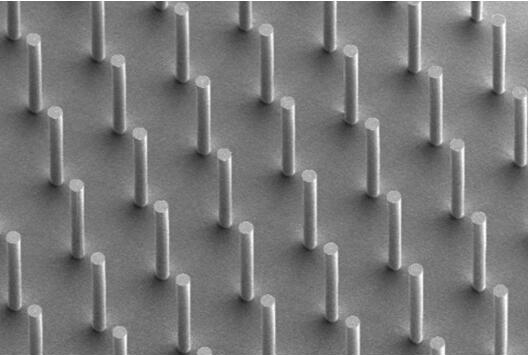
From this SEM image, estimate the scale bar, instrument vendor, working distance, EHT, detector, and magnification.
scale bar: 100000 nm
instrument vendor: Hitachi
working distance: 23.2 mm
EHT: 2 kV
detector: SE(L)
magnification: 0.5 K X